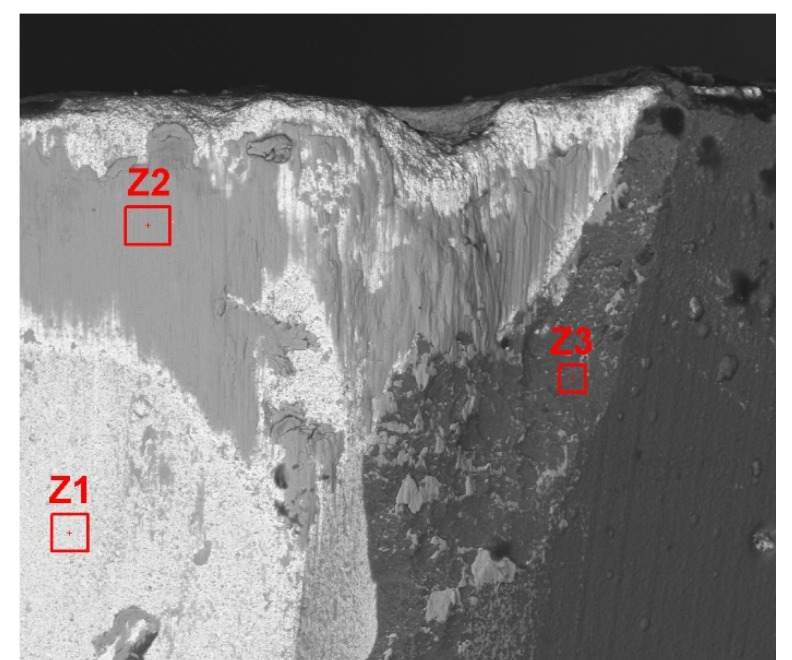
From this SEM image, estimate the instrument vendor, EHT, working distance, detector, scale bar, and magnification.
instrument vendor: FEI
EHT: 15 kV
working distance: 18.2 mm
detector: BSED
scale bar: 100000 nm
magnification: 1 K X